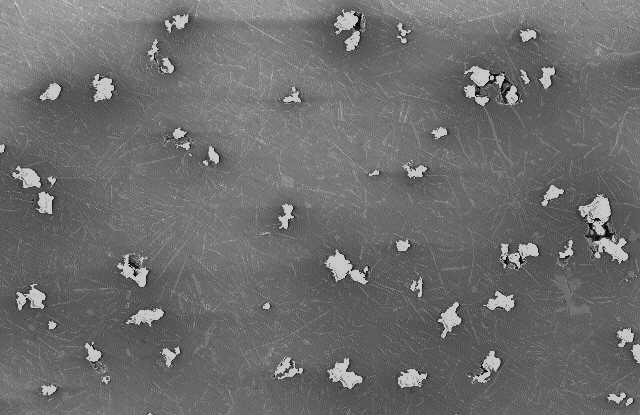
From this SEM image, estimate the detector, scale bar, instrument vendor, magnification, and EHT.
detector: SEI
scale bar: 500000 nm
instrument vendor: JEOL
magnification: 0.05 K X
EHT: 20 kV